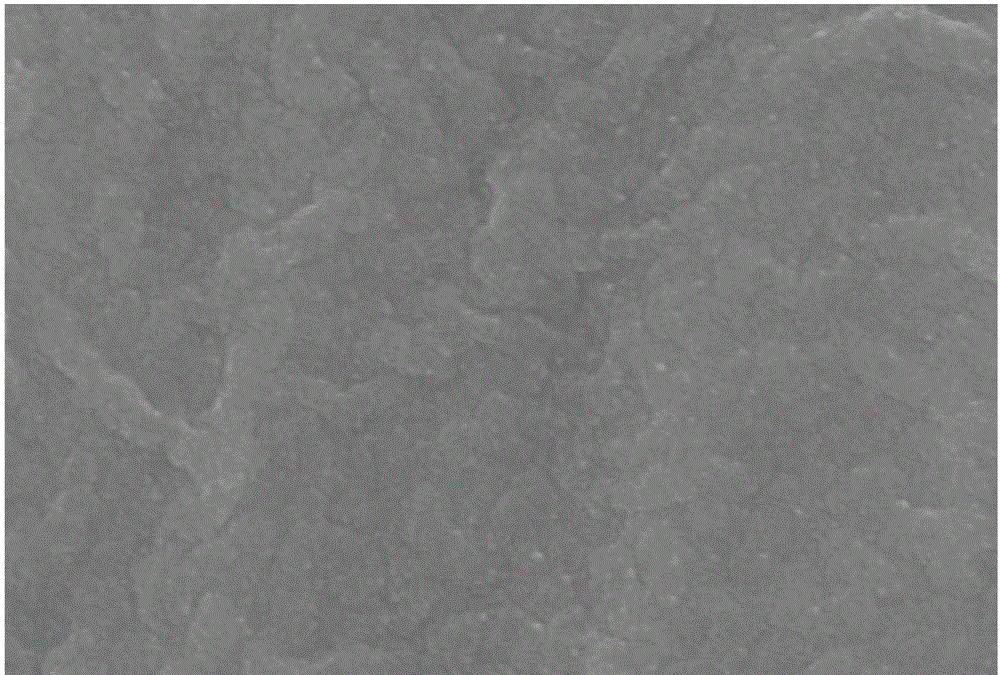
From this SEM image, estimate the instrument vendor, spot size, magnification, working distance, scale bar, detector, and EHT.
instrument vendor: JEOL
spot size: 30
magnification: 20 K X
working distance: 12 mm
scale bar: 1000 nm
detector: SEI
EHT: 20 kV